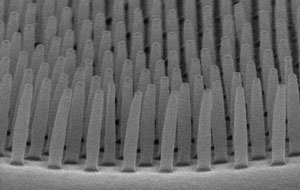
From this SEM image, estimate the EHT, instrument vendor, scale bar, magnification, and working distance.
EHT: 15 kV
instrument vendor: Hitachi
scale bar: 1000 nm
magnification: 40 K X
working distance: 11.3 mm